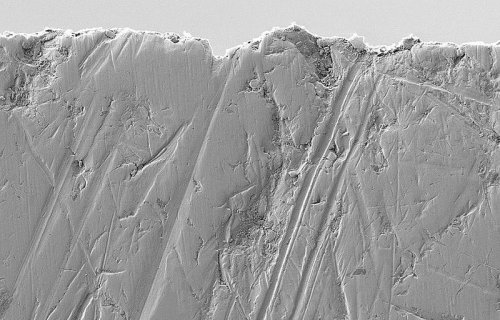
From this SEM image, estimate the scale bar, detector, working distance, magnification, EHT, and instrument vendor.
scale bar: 1000 nm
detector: SE2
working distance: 5.2 mm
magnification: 5 K X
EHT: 1 kV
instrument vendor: Zeiss